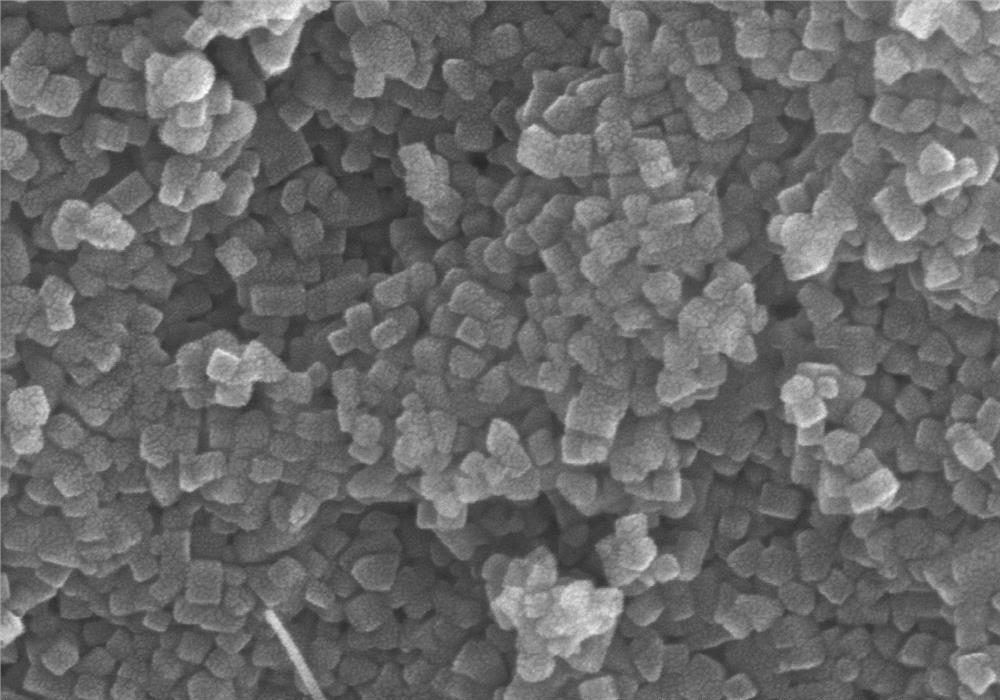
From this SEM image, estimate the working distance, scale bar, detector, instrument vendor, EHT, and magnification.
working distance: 9.7 mm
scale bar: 500 nm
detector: SE(M)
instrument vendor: Hitachi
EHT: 15 kV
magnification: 100 K X